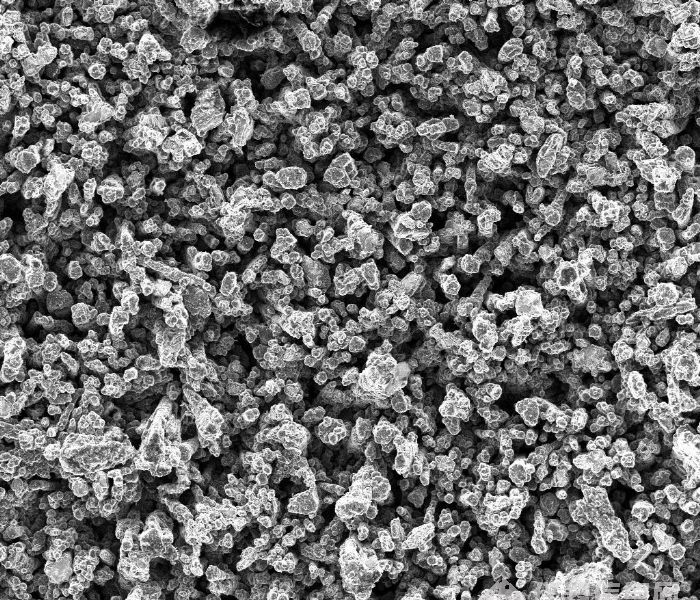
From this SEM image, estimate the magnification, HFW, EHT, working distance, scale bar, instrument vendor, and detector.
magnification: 1 K X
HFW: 240 µm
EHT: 5 kV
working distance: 6.5 mm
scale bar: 50000 nm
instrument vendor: FEI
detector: ETD